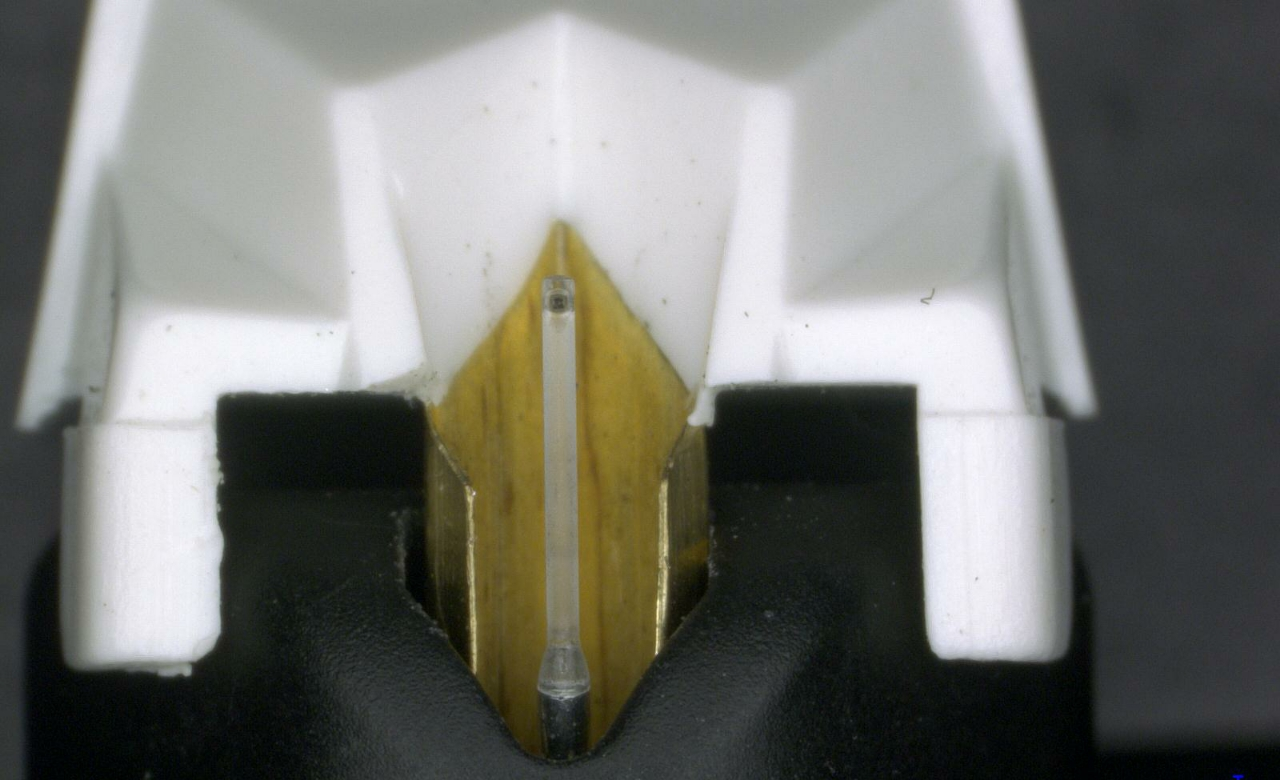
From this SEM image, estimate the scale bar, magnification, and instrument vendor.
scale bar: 1000000 nm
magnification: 0.03 K X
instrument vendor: other